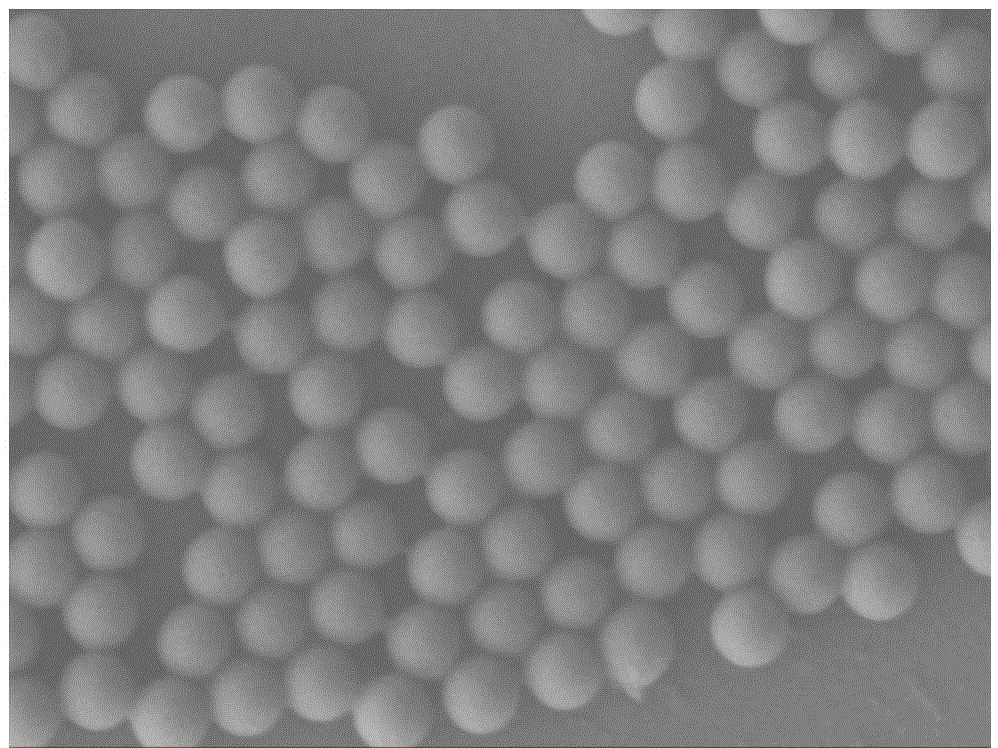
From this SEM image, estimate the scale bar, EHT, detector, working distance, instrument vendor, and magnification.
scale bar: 100 nm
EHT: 3 kV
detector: LEI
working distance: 7 mm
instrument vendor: JEOL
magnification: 30 K X